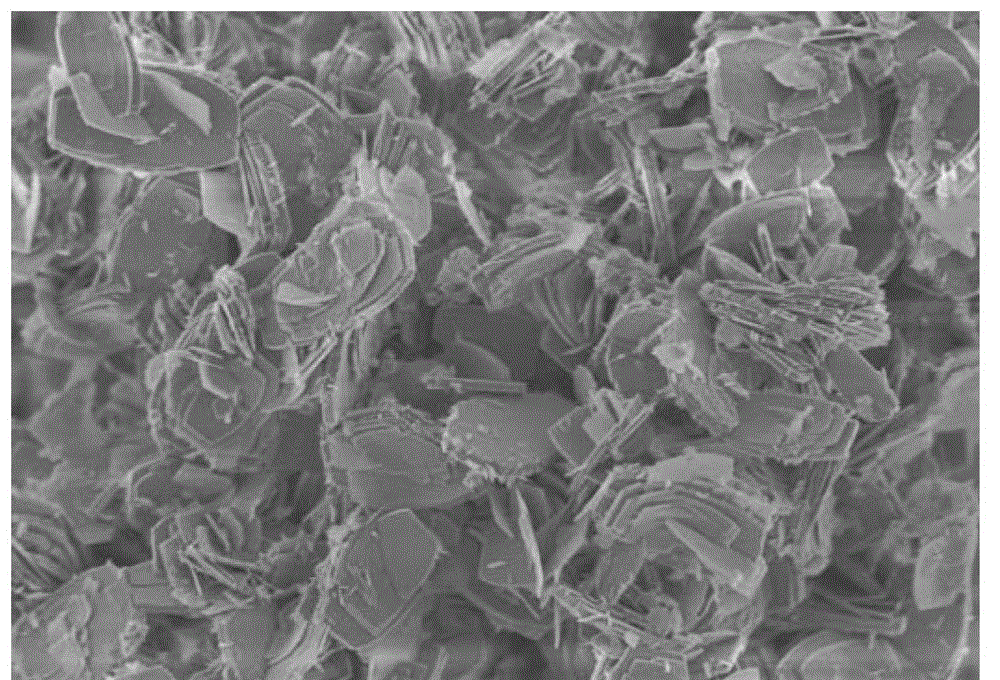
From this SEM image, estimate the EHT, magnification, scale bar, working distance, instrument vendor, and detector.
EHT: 2 kV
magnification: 5 K X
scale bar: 10000 nm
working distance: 6.2 mm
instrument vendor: Hitachi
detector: SE+BSE(U)